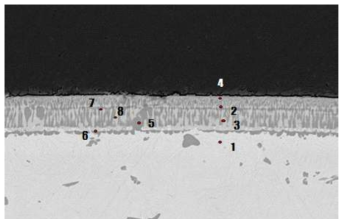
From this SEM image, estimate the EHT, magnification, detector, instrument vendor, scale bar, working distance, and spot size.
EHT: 15 kV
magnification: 1.5 K X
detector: BSE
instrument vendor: FEI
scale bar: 20000 nm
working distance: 10.2 mm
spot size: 5